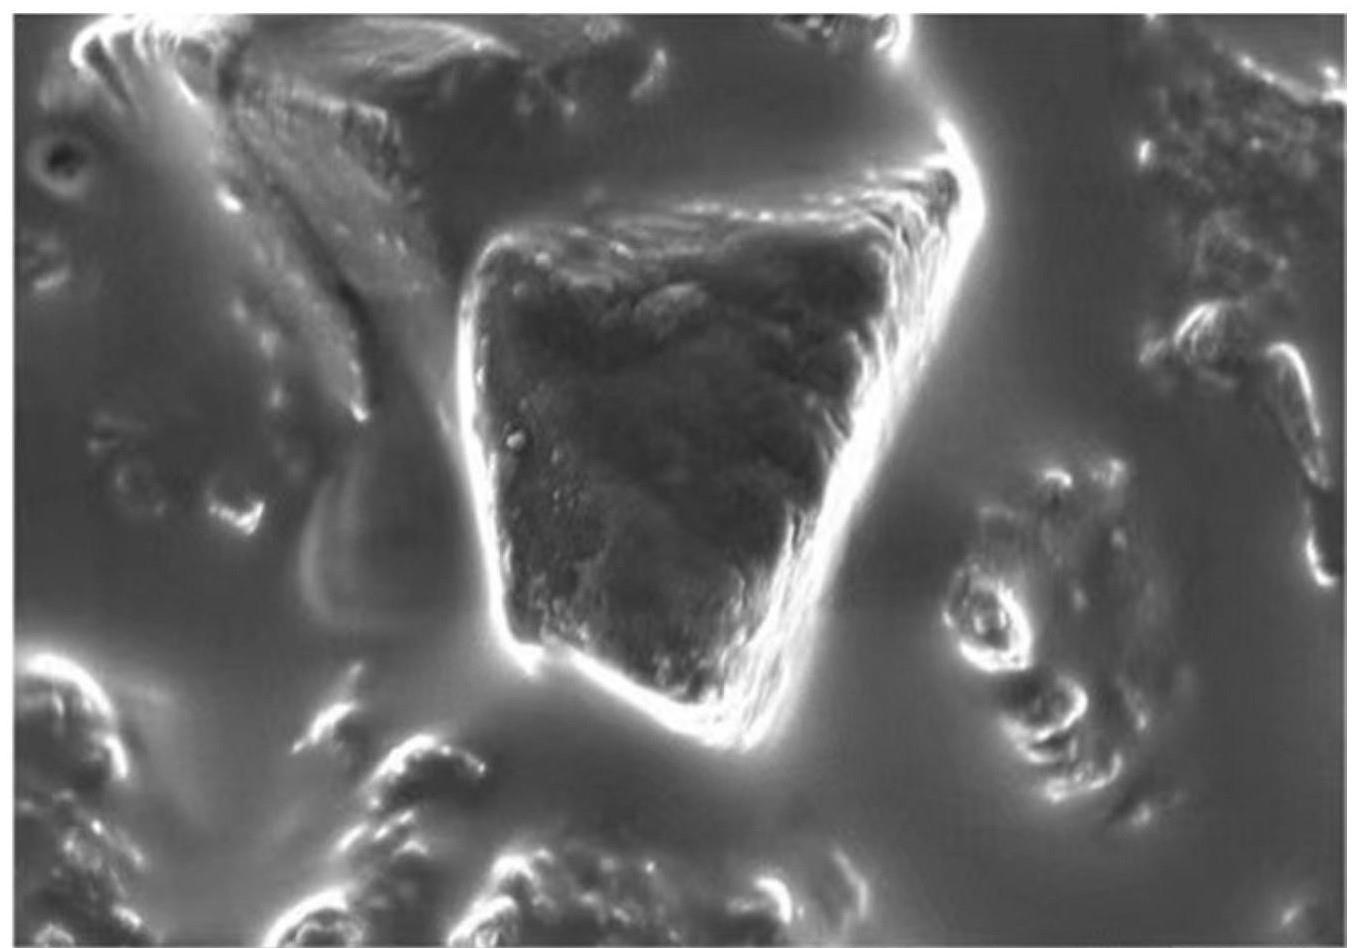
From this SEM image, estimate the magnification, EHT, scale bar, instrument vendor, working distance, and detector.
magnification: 10 K X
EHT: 5 kV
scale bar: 2000 nm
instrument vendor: Zeiss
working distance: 4.9 mm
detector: InlensDuo